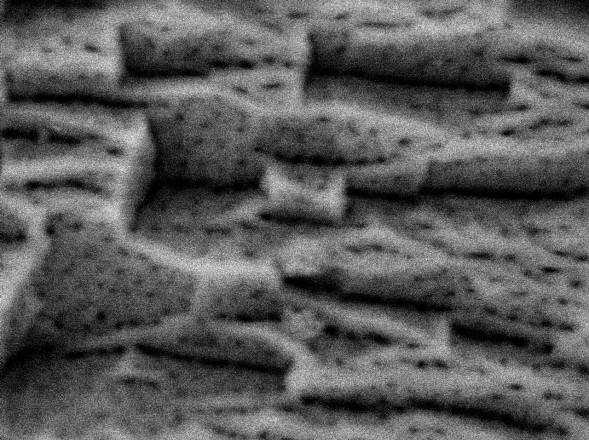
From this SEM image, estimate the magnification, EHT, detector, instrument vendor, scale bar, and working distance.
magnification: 60 K X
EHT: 5 kV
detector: AUX1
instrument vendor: JEOL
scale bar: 100 nm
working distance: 9.2 mm